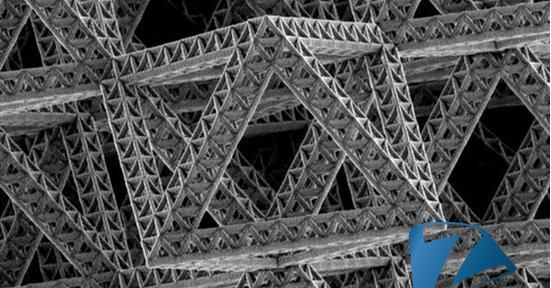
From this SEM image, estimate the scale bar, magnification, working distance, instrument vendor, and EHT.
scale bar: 10000 nm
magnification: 2.048 K X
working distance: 4.8 mm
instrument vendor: FEI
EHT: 5 kV